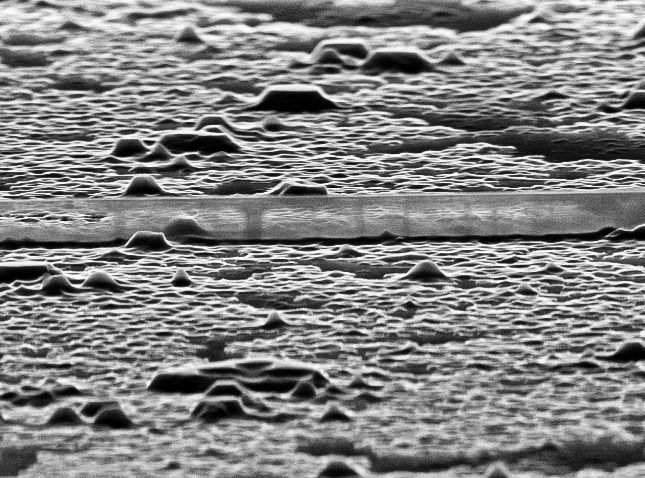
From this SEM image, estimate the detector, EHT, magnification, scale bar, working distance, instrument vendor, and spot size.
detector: SE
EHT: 30 kV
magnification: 16.119 K X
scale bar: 1000 nm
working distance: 15.4 mm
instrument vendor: FEI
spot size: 4